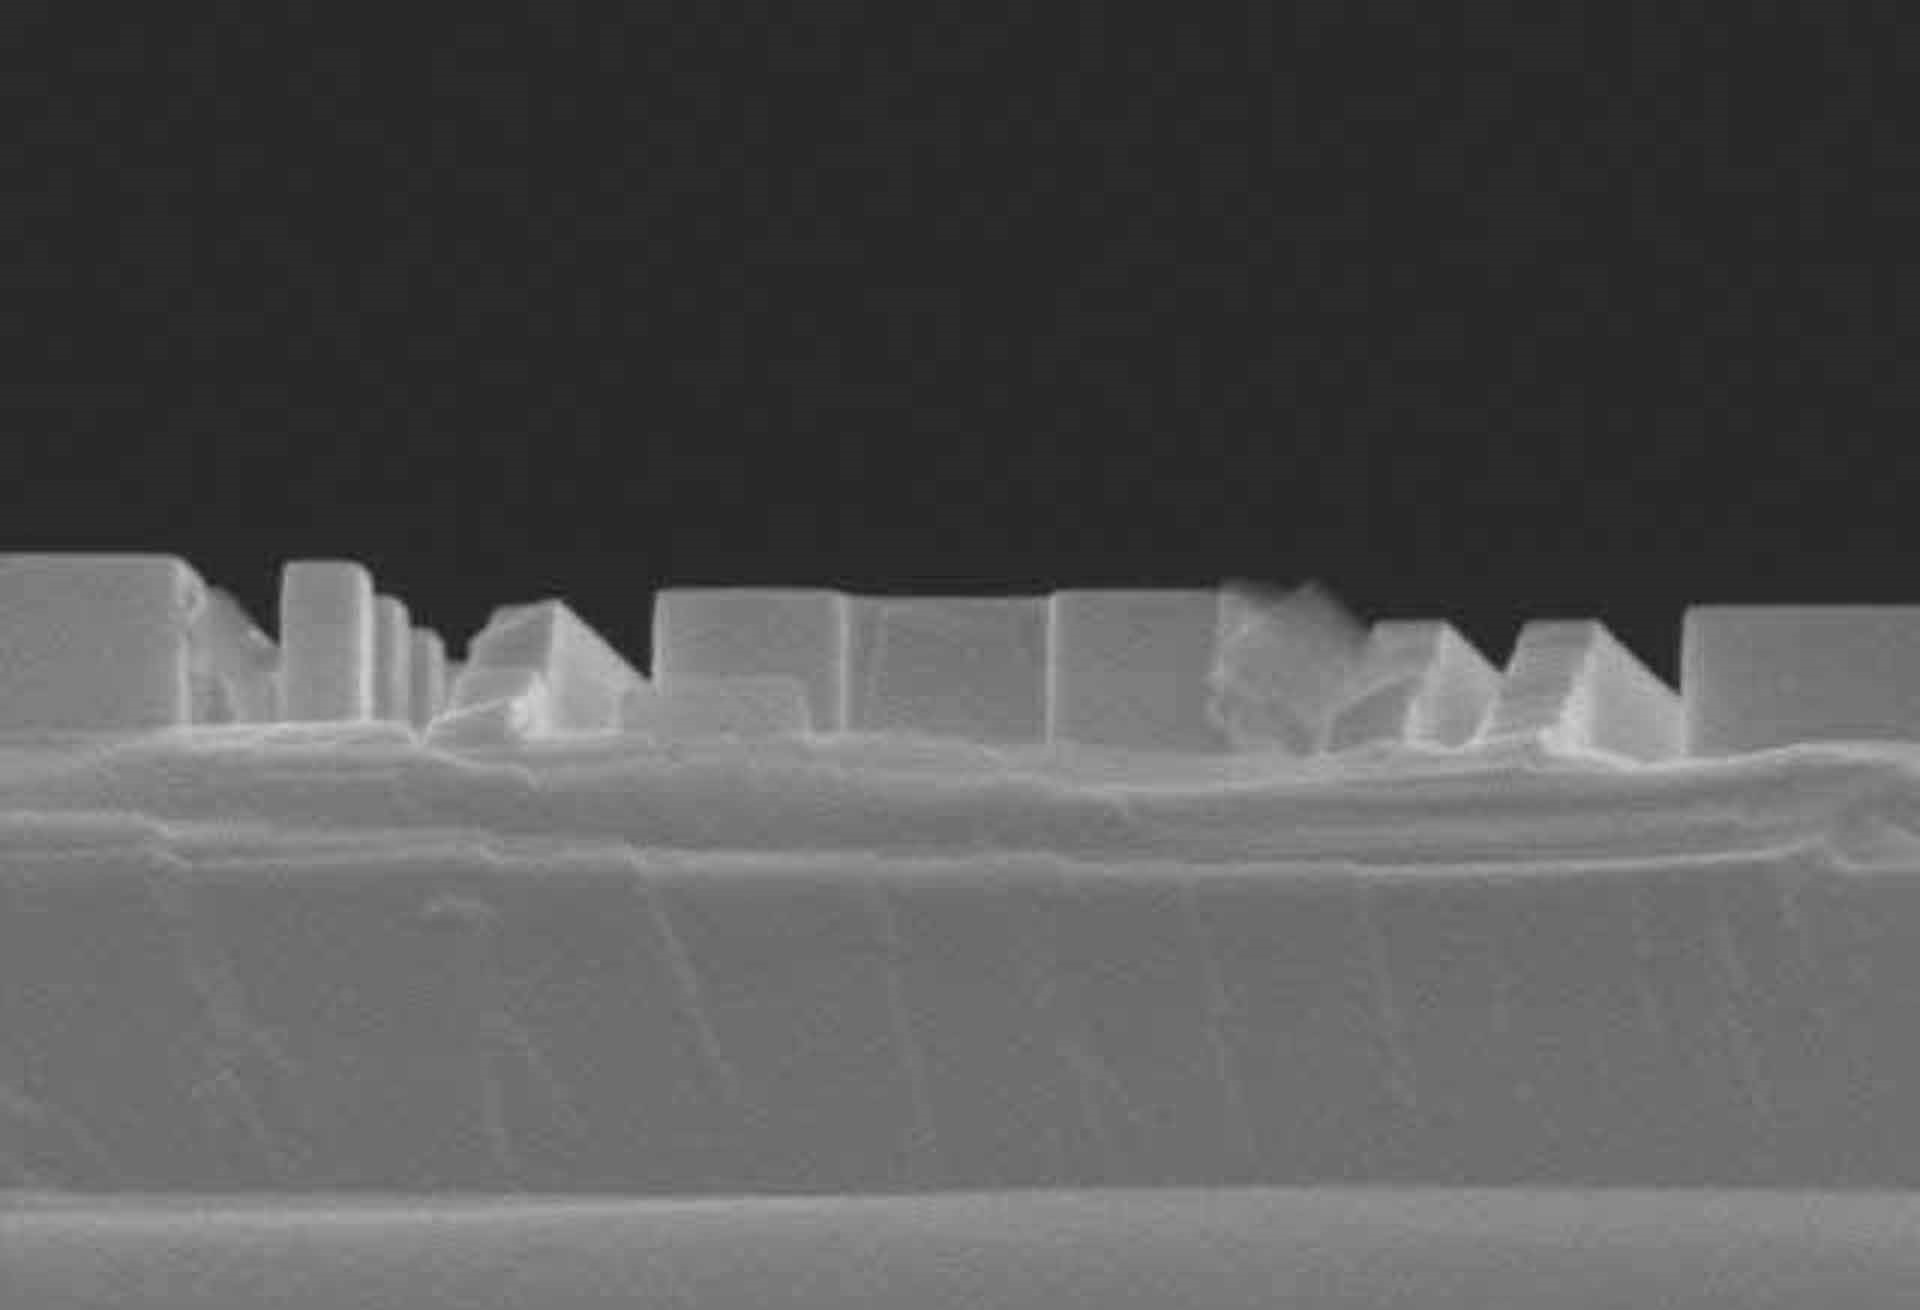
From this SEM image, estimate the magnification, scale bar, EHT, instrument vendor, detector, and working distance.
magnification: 40 K X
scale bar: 1000 nm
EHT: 15 kV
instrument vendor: Hitachi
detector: SE(M)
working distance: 15.8 mm